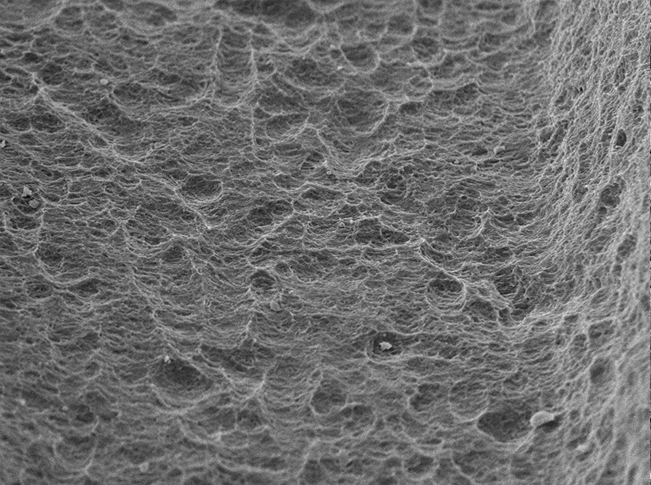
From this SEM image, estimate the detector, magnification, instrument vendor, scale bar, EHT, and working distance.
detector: SE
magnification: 1 K X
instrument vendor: other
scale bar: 50000 nm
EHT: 15 kV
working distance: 6.9 mm